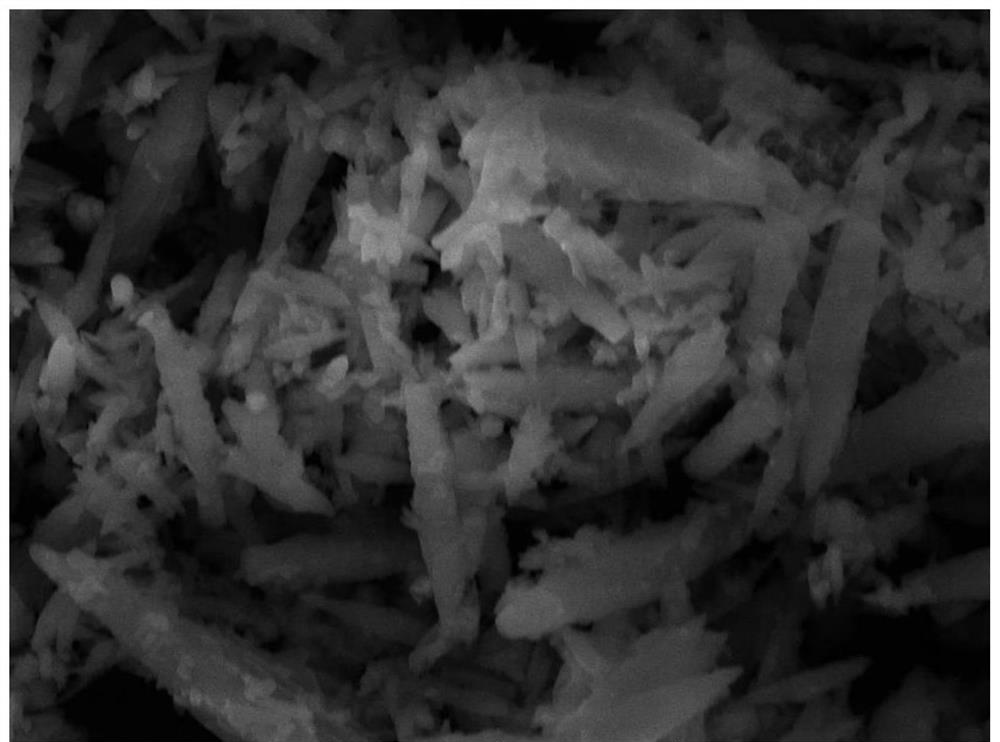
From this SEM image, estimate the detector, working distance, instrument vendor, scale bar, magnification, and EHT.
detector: SE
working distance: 10.13 mm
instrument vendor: Tescan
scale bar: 10000 nm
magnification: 5 K X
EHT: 20 kV